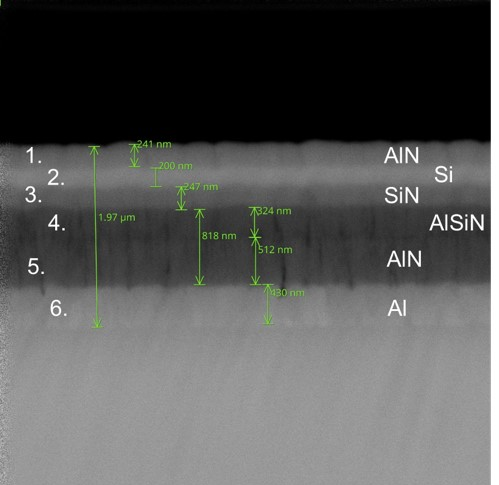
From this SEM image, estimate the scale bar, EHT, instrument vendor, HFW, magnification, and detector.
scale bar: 1000 nm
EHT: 10 kV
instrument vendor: other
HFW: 5.37 µm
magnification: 50 K X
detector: BSD Full